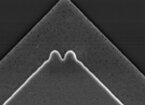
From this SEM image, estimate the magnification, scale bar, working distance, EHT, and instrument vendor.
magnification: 10 K X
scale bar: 2000 nm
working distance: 5 mm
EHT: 12 kV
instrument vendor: FEI